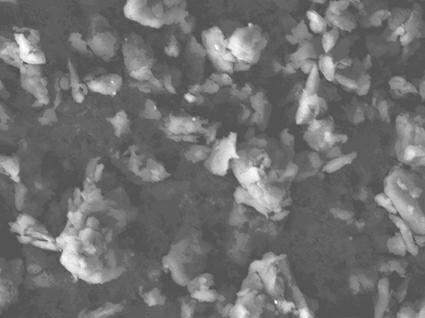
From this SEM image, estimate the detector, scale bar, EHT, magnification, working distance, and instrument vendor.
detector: LEI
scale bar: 1000 nm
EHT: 10 kV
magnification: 3 K X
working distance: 8.1 mm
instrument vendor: JEOL